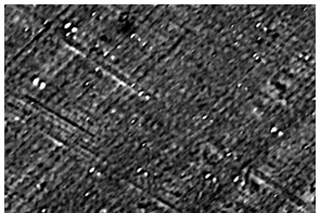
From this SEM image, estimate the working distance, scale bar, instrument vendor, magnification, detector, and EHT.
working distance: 10 mm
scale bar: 10000 nm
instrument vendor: JEOL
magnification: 2.5 K X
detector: SED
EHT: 10 kV